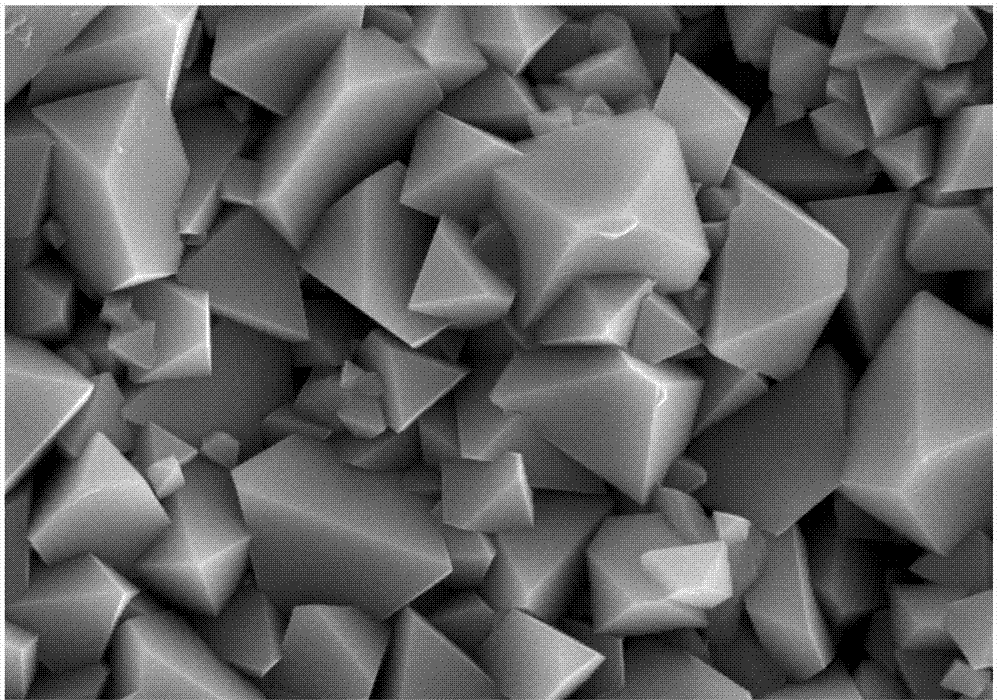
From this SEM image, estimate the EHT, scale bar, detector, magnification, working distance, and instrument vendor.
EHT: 10 kV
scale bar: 200 nm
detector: SE2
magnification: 20 K X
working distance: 11.1 mm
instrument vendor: Zeiss